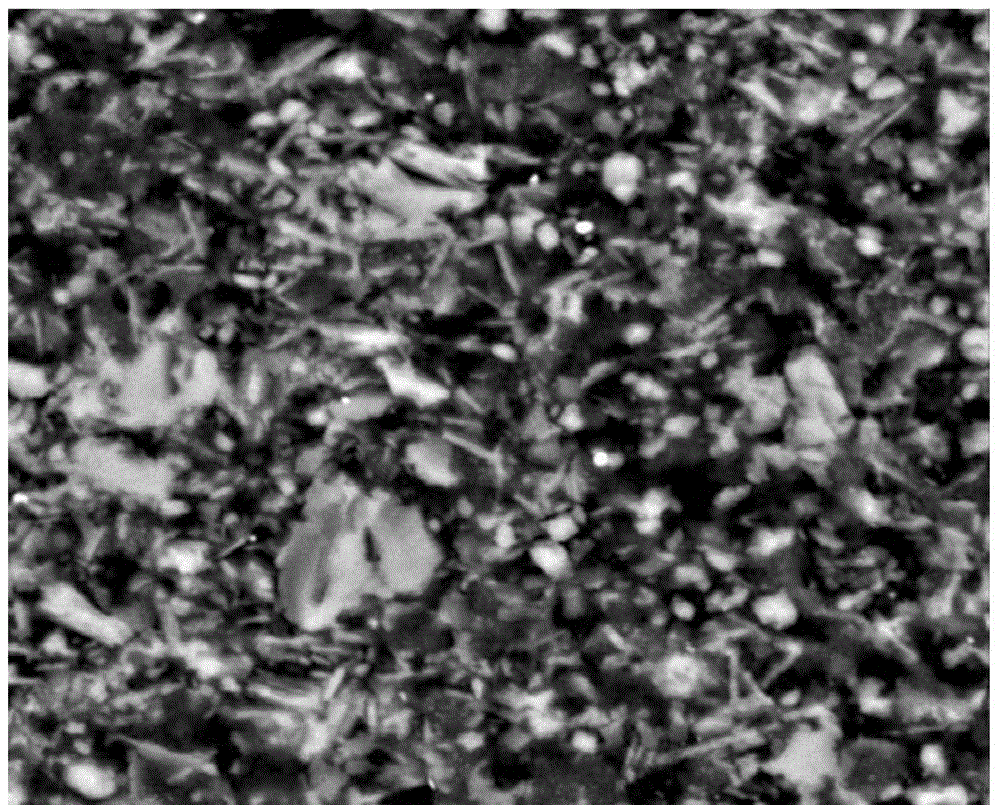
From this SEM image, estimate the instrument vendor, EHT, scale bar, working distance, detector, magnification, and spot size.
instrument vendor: FEI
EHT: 20 kV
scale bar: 20000 nm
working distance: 10.3 mm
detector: SSD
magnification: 2.5 K X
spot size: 4.5